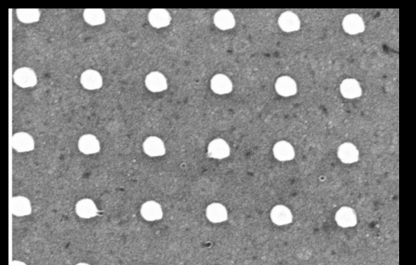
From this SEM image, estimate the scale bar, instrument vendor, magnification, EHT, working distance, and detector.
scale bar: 200 nm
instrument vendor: Zeiss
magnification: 32.65 K X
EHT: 20 kV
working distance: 10 mm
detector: InLens